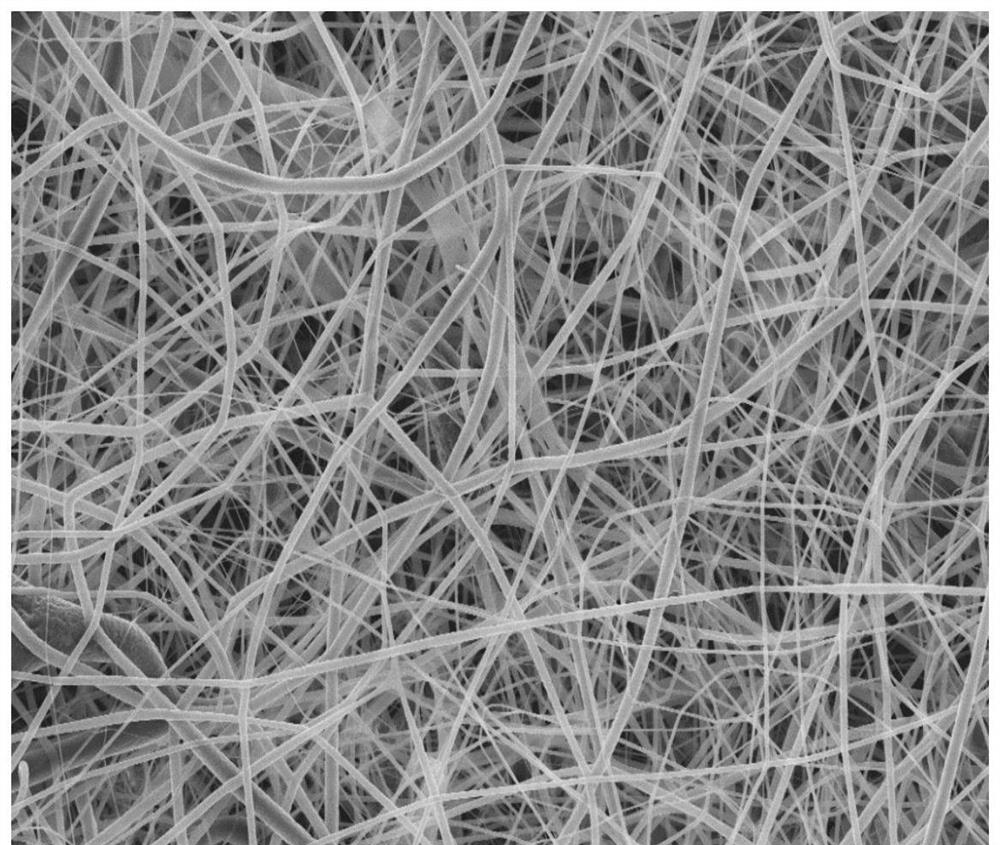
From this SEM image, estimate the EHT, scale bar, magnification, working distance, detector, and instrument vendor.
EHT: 10 kV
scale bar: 10000 nm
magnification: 1 K X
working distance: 8.5 mm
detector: InLens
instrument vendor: Zeiss